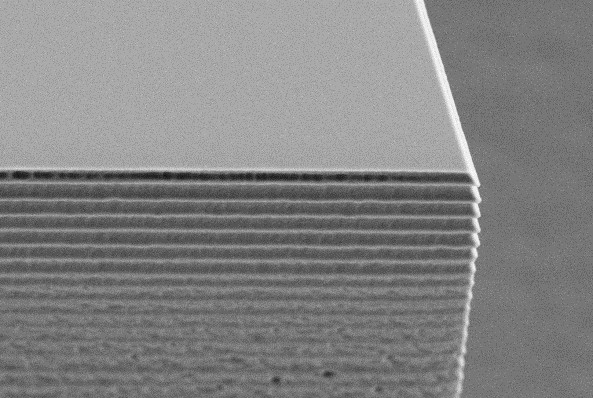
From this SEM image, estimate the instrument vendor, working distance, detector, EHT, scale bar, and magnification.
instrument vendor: Zeiss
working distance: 4.9 mm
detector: SE2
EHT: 5 kV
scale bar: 1000 nm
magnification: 10 K X